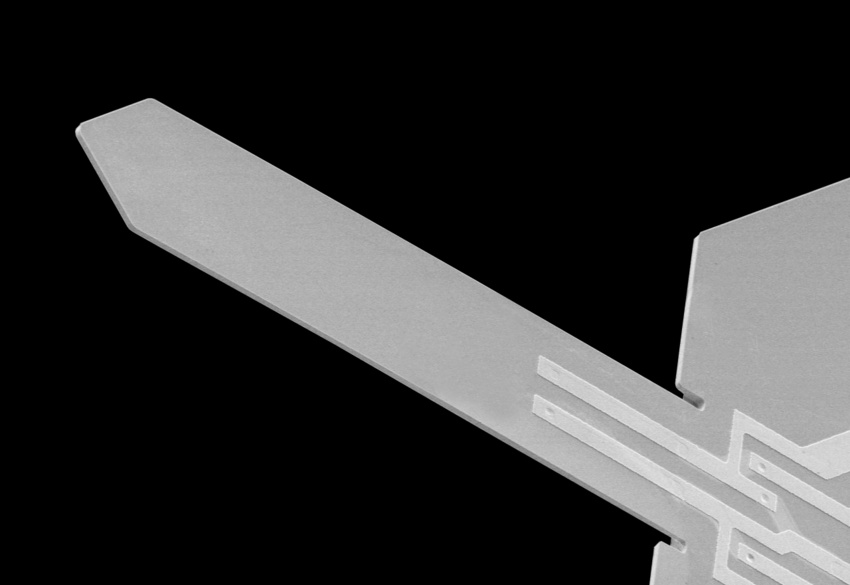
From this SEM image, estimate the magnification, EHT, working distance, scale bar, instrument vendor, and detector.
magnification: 0.25 K X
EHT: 4 kV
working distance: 9.9 mm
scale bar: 200000 nm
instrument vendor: Hitachi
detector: LM(UL)